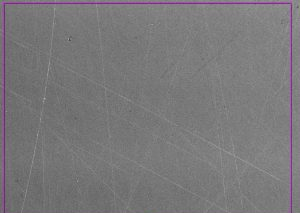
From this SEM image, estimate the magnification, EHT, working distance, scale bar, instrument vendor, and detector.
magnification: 0.25 K X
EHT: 20 kV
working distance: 10 mm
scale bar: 100000 nm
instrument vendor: other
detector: SED